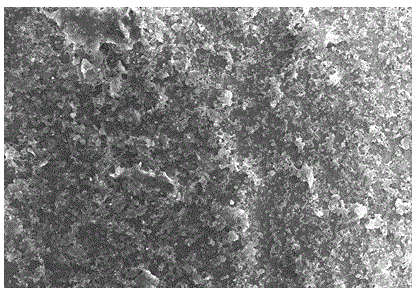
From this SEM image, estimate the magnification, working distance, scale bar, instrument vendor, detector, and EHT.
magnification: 1 K X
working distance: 7.6 mm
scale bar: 50000 nm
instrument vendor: Hitachi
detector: SE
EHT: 15 kV